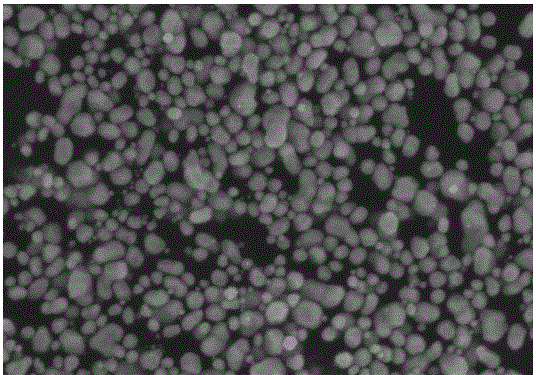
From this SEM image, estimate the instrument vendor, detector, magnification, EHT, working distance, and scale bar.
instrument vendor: Hitachi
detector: SE(U)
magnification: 45 K X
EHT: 5 kV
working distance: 6.3 mm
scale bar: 1000 nm